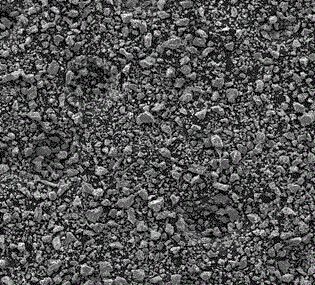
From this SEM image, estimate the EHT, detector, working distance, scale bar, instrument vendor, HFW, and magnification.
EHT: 30 kV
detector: SE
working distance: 20.25 mm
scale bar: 200000 nm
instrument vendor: Tescan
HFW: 1440 µm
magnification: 0.1 K X